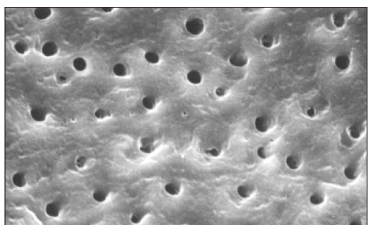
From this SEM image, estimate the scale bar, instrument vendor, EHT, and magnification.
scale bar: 20000 nm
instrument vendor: Hitachi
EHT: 20 kV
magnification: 2 K X